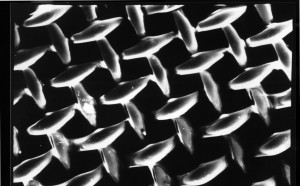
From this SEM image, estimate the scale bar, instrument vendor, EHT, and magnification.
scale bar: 100000 nm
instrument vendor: JEOL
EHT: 10 kV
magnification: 0.2 K X